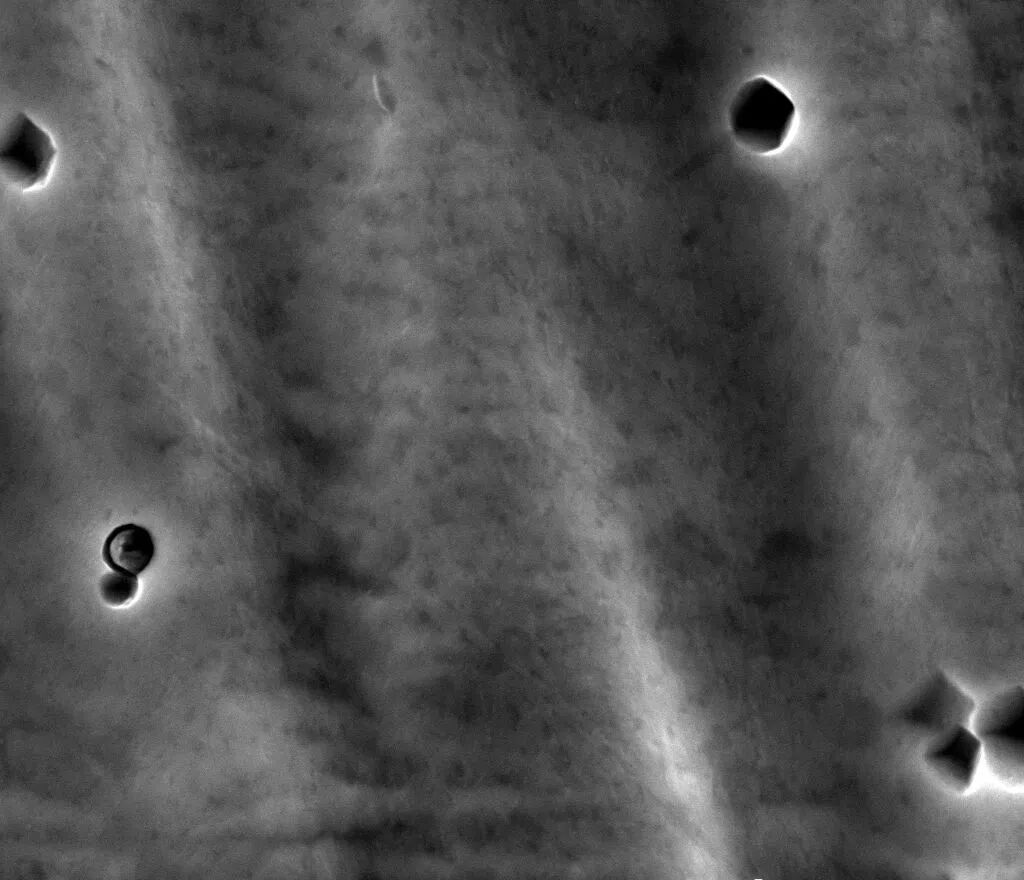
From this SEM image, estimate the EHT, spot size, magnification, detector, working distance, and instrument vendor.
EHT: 20 kV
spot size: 4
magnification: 10 K X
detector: ETD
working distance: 8 mm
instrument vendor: FEI